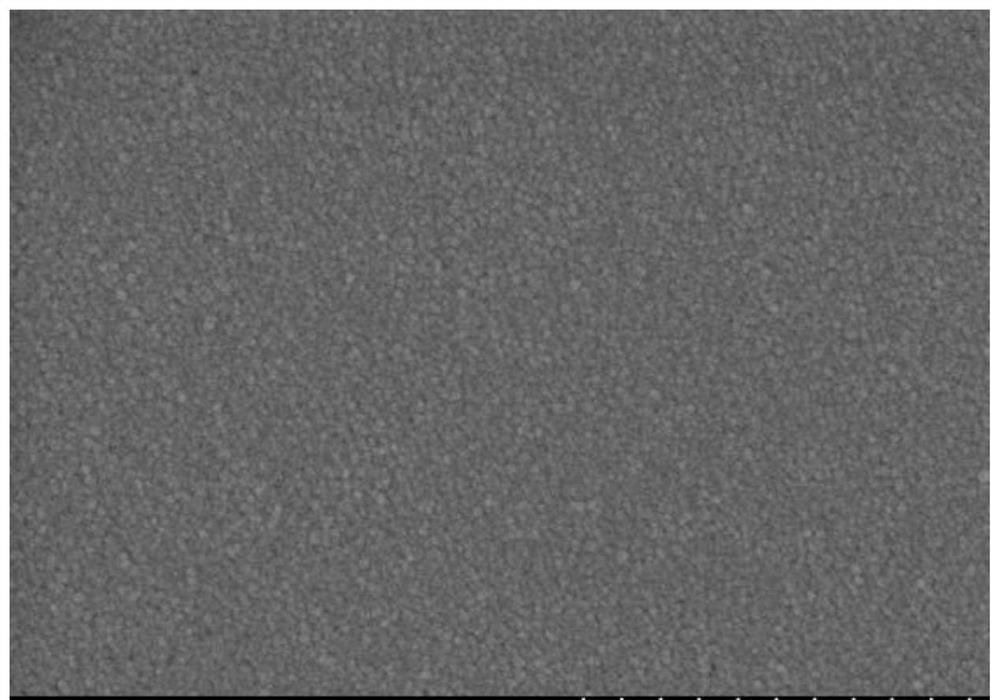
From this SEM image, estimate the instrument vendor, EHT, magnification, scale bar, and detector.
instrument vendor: Hitachi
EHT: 4 kV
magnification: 100 K X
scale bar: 500 nm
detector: SE(M)